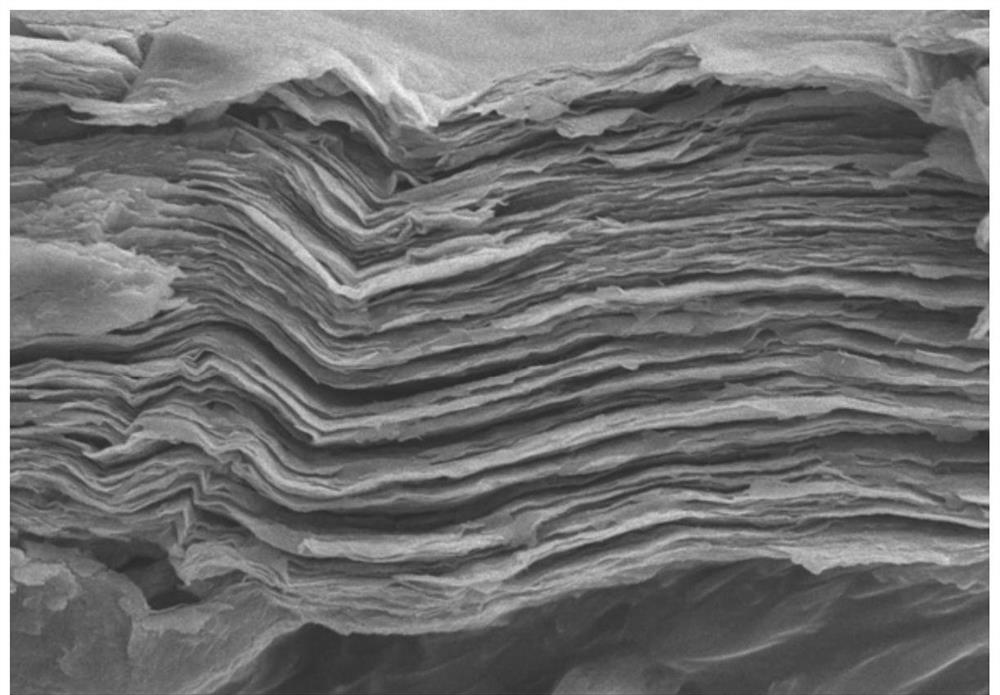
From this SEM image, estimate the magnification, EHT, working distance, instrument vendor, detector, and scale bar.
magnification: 15 K X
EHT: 5 kV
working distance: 9.6 mm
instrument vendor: Hitachi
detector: SE(M)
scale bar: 3000 nm